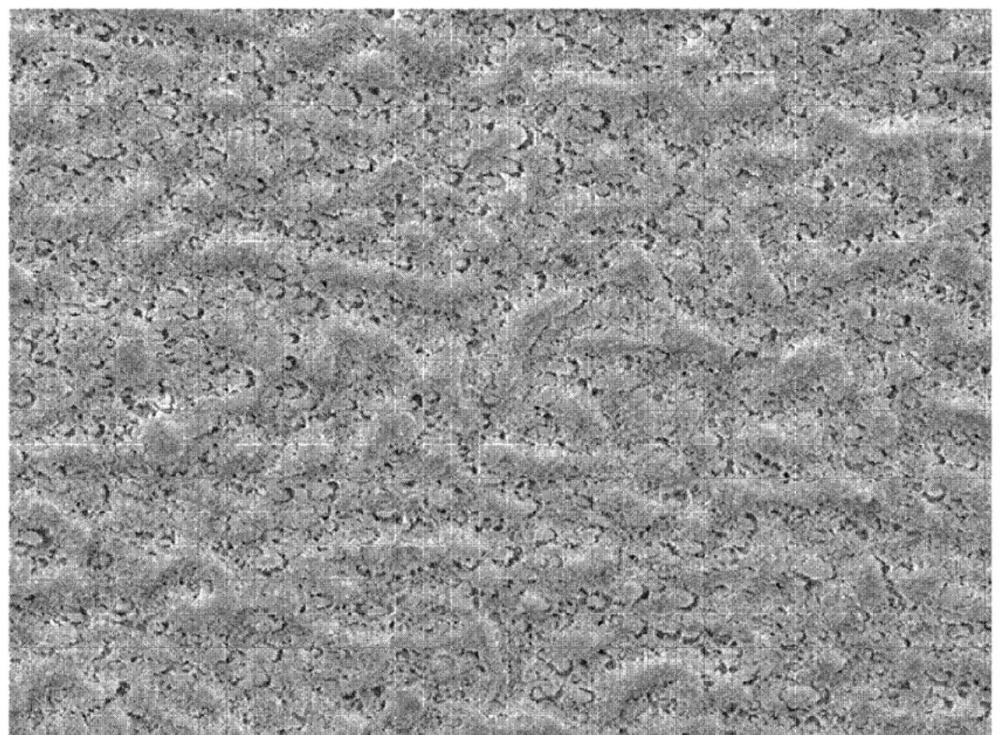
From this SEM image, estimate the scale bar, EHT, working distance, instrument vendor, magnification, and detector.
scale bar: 10000 nm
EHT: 5 kV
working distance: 10 mm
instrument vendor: JEOL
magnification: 1 K X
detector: LED